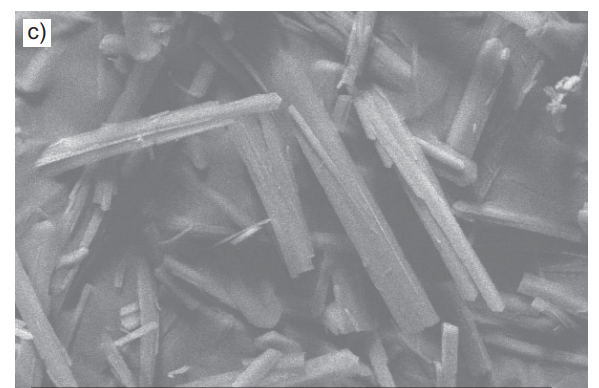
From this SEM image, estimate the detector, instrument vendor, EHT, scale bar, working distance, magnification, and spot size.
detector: SE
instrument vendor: FEI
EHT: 20 kV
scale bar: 50000 nm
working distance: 14.7 mm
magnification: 0.5 K X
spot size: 5.7